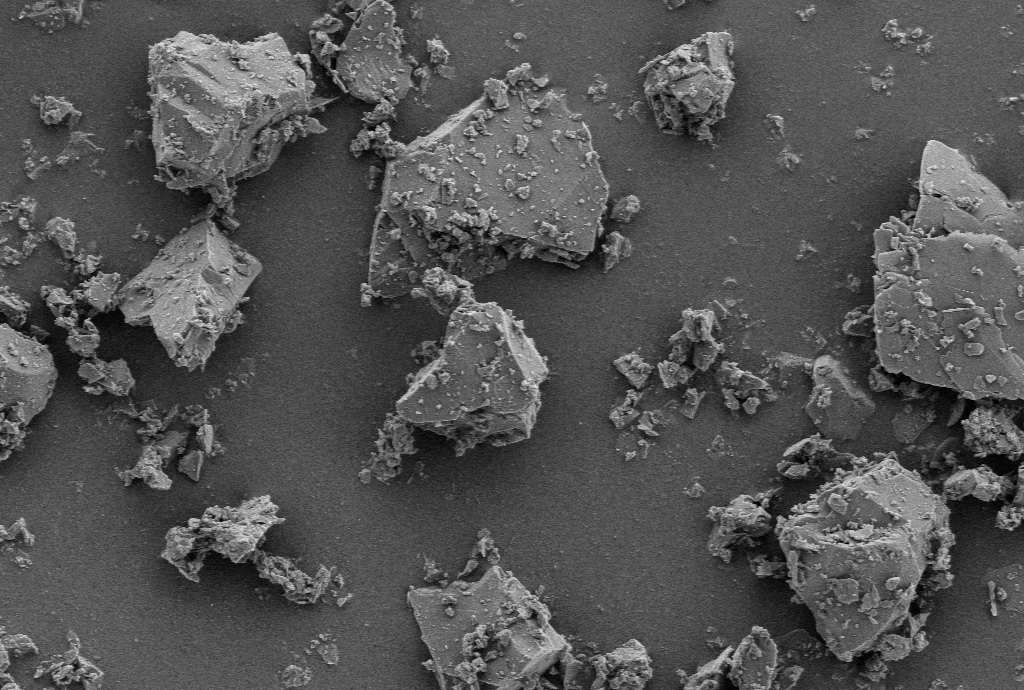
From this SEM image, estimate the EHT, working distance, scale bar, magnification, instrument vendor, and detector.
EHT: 3 kV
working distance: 7.9 mm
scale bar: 5000 nm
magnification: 5 K X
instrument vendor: Zeiss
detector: SE2